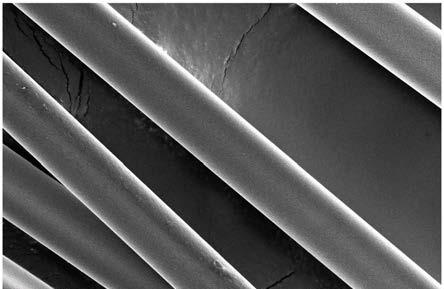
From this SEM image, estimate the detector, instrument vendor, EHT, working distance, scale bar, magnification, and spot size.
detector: T2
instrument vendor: FEI/Thermo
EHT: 10 kV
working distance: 9.5 mm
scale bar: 50000 nm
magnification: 1 K X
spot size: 7